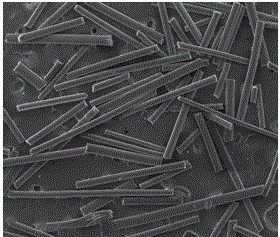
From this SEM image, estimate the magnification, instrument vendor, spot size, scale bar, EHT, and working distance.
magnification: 0.09 K X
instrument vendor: FEI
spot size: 6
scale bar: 1000000 nm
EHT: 20 kV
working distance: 11.3 mm